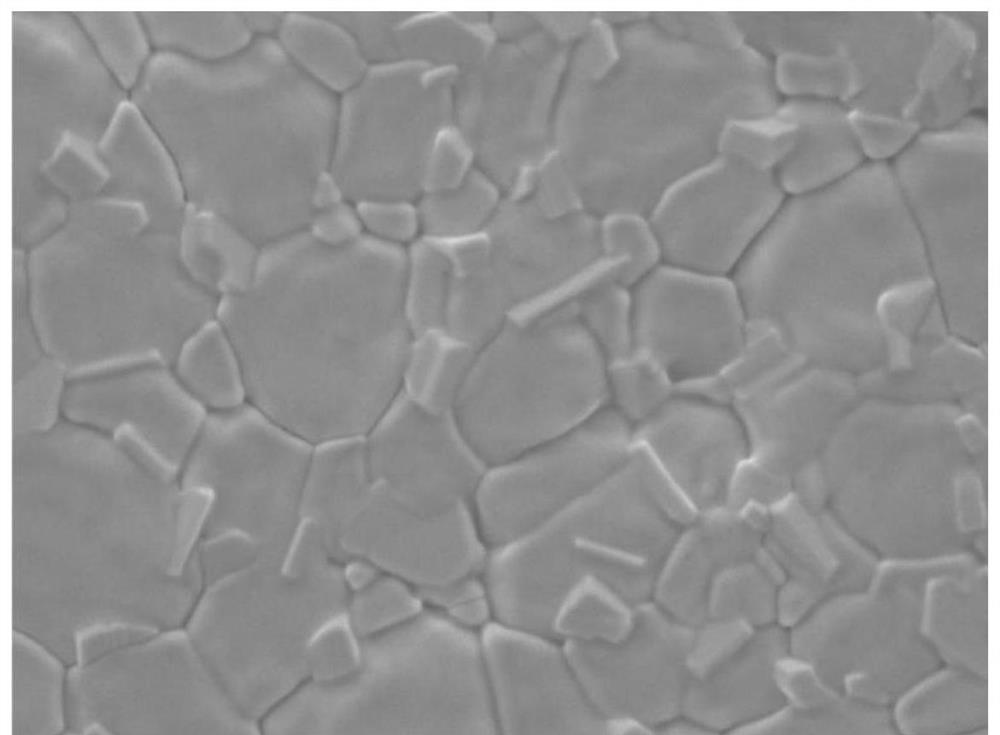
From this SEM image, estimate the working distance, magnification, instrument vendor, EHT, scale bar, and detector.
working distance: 12 mm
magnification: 15 K X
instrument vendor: JEOL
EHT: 3 kV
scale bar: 1000 nm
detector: LED